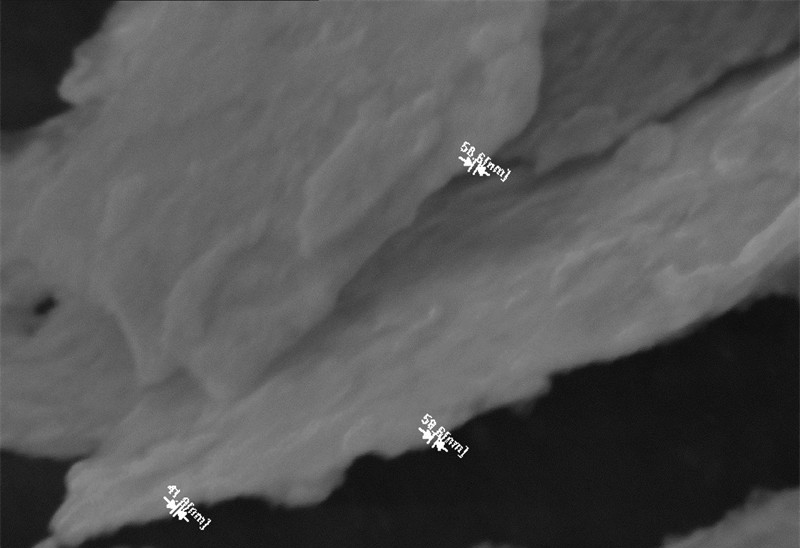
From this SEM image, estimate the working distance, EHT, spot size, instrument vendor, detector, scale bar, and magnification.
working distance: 8.9 mm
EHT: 10 kV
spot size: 10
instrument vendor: JEOL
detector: SEI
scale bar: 1000 nm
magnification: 30 K X